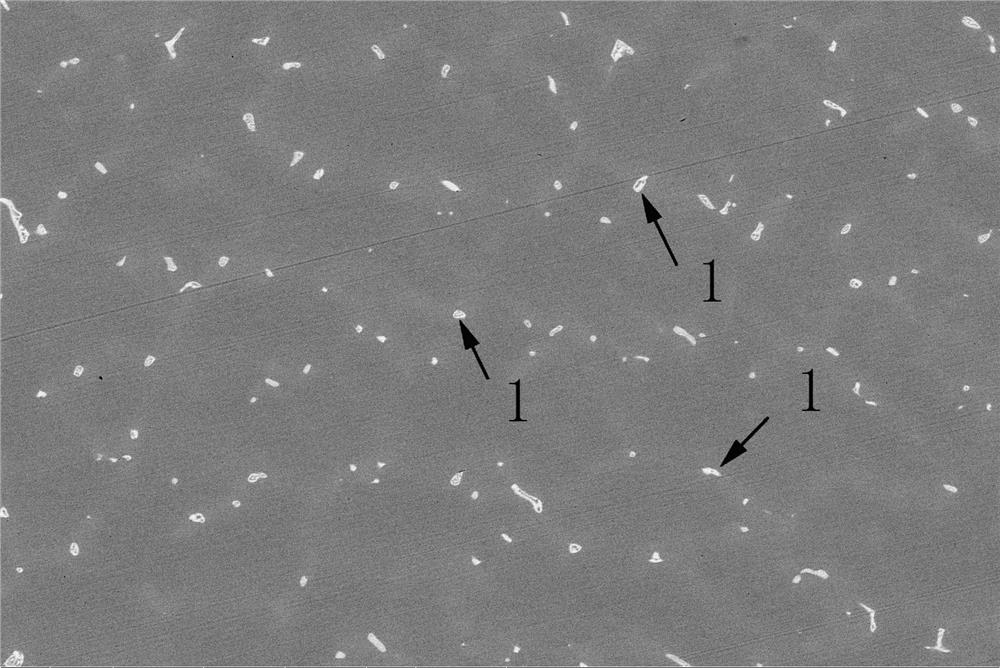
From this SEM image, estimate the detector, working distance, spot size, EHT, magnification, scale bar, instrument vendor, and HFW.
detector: BSED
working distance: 13.3 mm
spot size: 3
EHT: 20 kV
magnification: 2 K X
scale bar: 50000 nm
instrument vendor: FEI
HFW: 207 µm